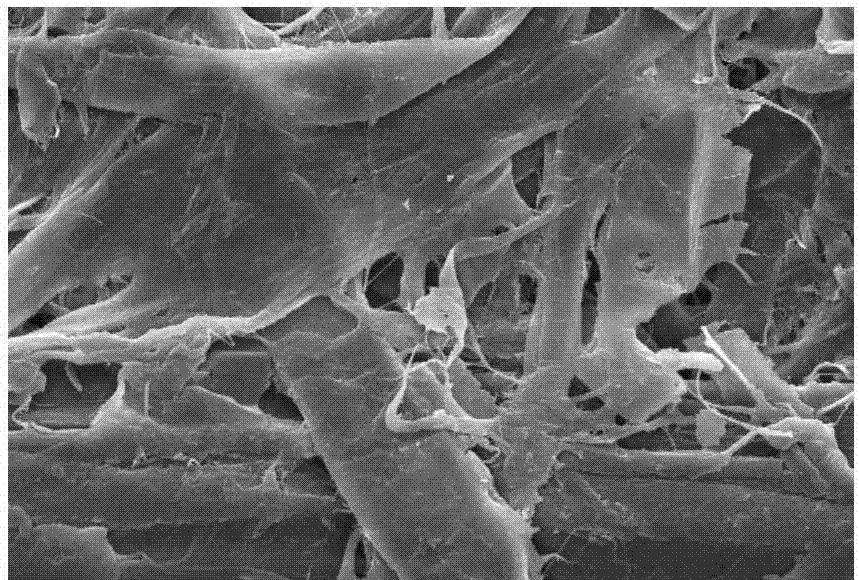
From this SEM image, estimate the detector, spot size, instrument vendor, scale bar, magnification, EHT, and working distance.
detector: SE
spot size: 3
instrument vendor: FEI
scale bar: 50000 nm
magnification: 0.5 K X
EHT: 20 kV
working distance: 6.2 mm